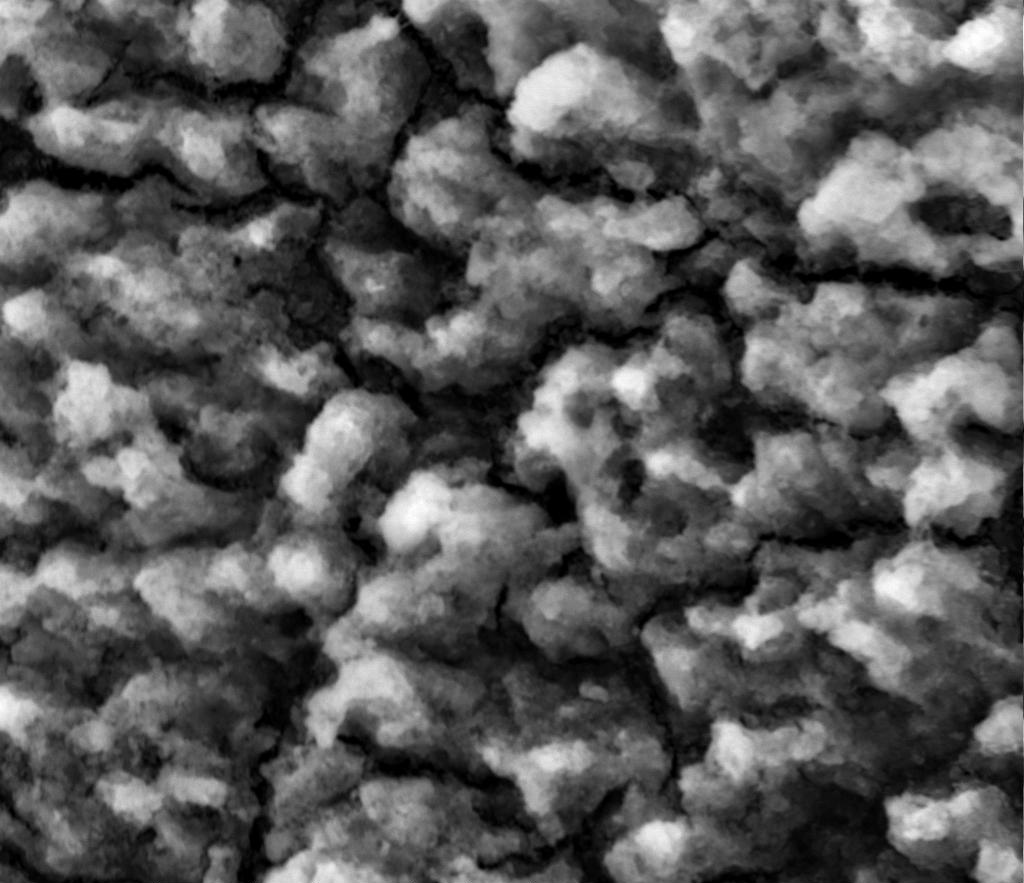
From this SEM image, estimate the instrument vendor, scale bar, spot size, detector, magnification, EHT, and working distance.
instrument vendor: FEI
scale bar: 100000 nm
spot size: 5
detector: Mix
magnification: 0.5 K X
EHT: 30 kV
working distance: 9.7 mm